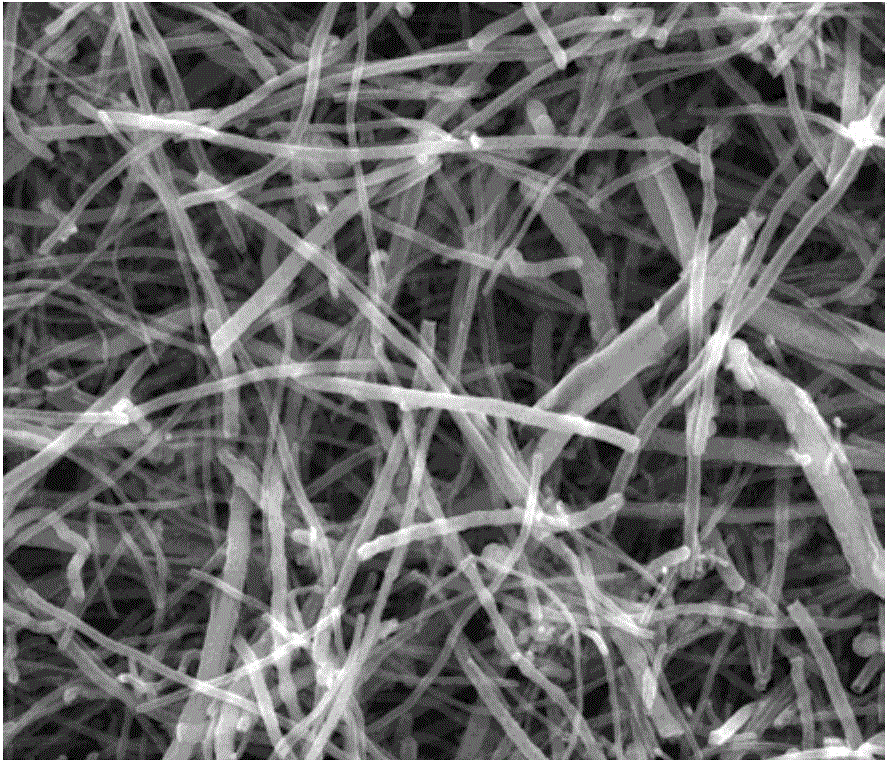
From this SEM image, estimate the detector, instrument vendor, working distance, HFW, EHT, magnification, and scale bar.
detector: ETD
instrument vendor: FEI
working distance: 11 mm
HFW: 14.9 µm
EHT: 10 kV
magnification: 20 K X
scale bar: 5000 nm